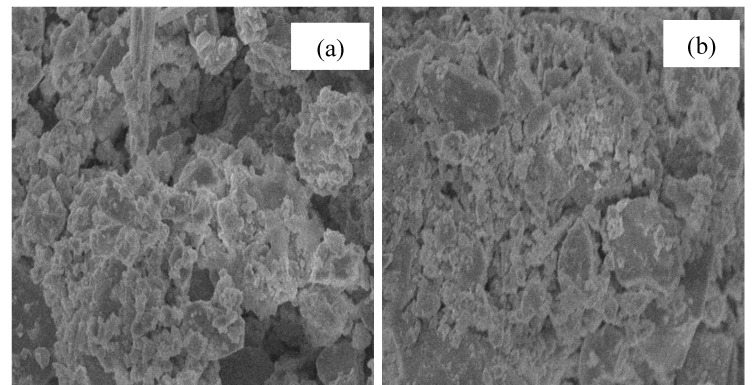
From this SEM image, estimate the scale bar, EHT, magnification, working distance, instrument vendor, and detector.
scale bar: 1000 nm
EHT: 8 kV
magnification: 5 K X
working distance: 8.7 mm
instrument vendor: JEOL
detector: SEI